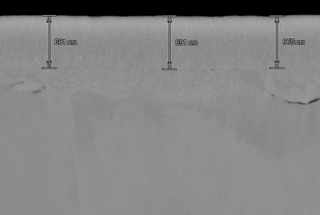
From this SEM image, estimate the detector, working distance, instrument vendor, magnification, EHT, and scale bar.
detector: LA50(UL)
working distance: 8 mm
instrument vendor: Hitachi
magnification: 30 K X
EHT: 10 kV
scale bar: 1000 nm